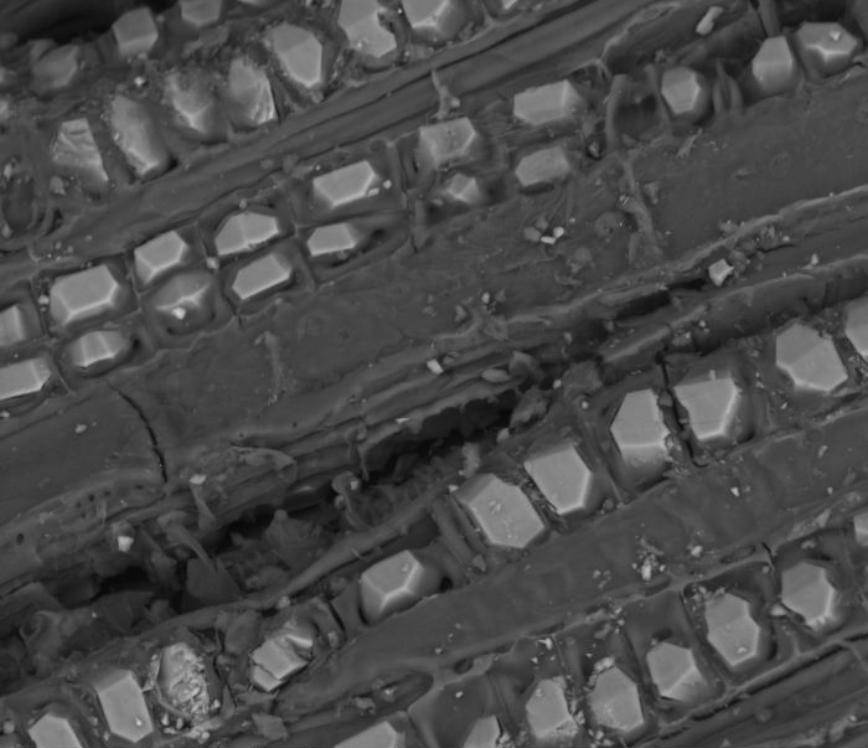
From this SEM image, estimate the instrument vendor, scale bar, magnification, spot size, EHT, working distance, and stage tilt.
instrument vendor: FEI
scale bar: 50000 nm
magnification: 1 K X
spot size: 4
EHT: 15 kV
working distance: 11.5 mm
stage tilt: -0.4°